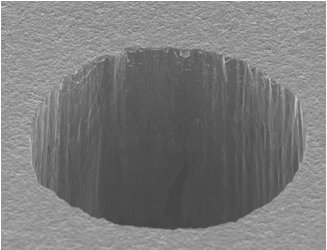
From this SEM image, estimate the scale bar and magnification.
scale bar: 100000 nm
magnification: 0.25 K X